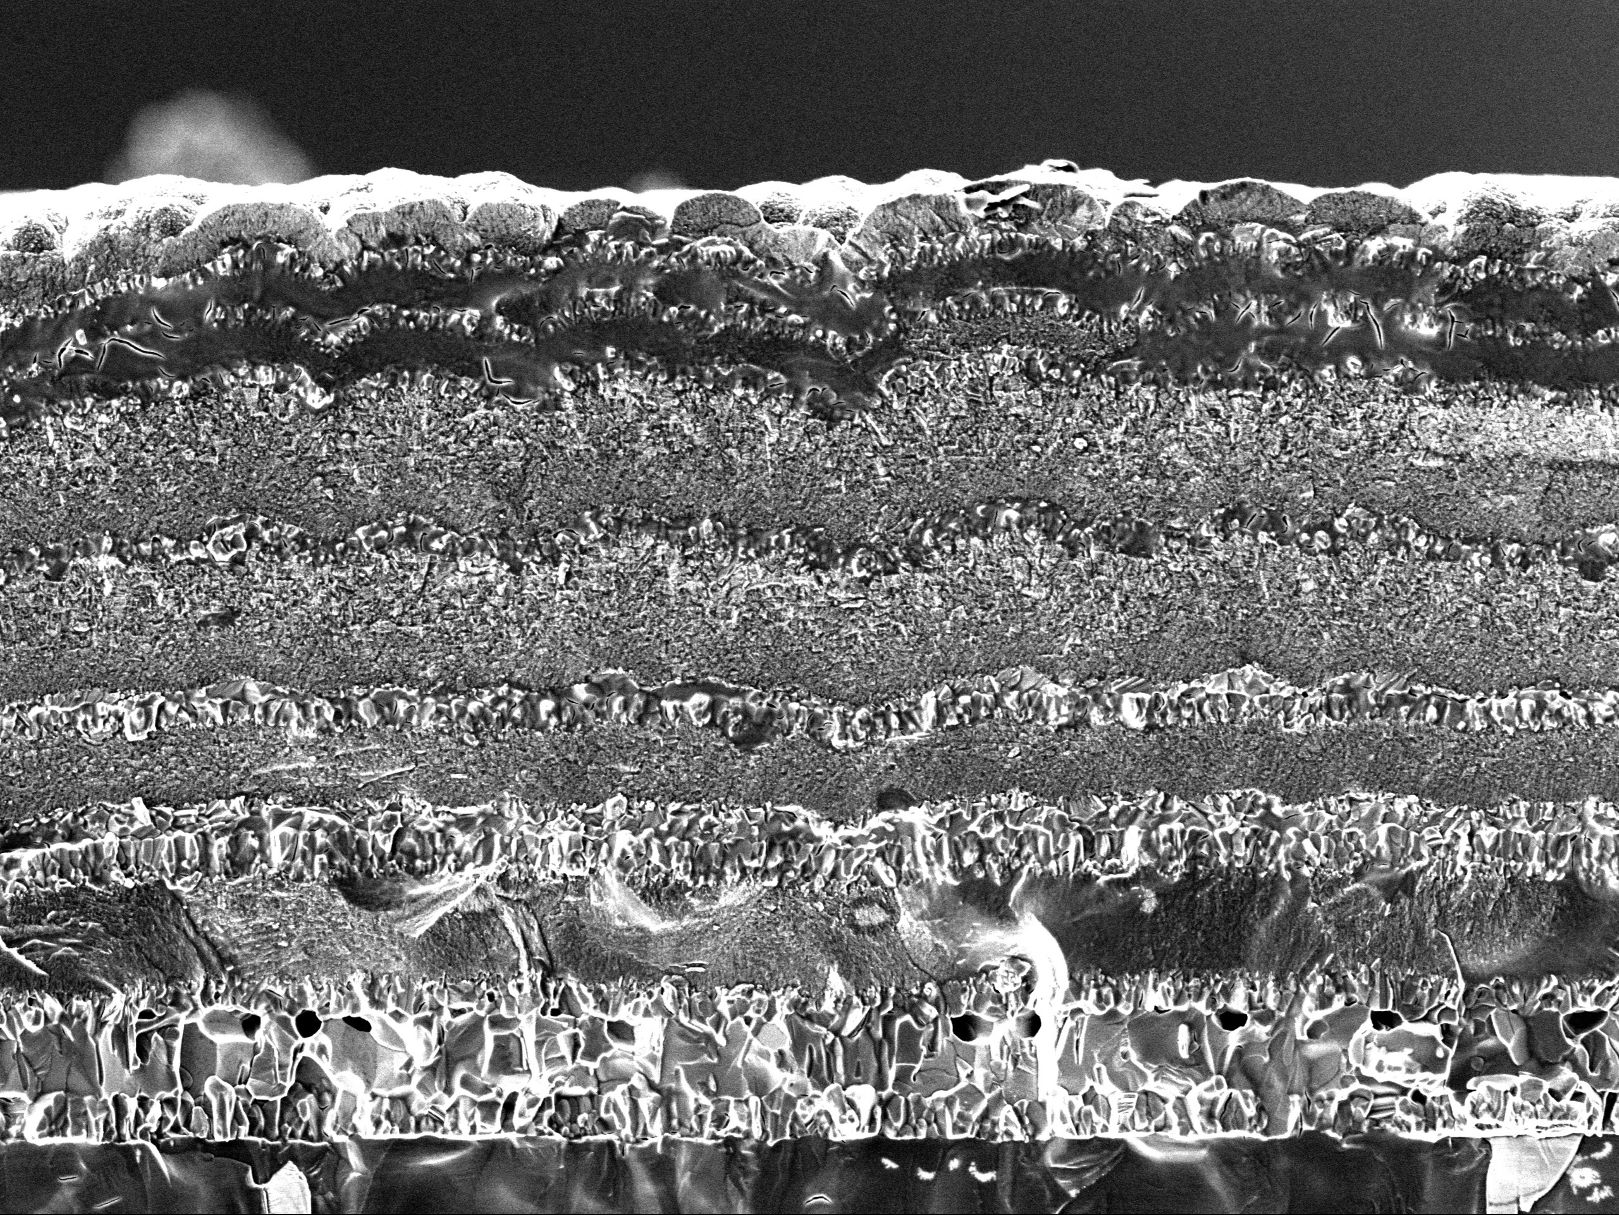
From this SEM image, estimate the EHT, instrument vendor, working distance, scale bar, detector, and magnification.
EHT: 3 kV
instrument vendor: JEOL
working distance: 9.8 mm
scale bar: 5000 nm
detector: SED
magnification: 2.7 K X